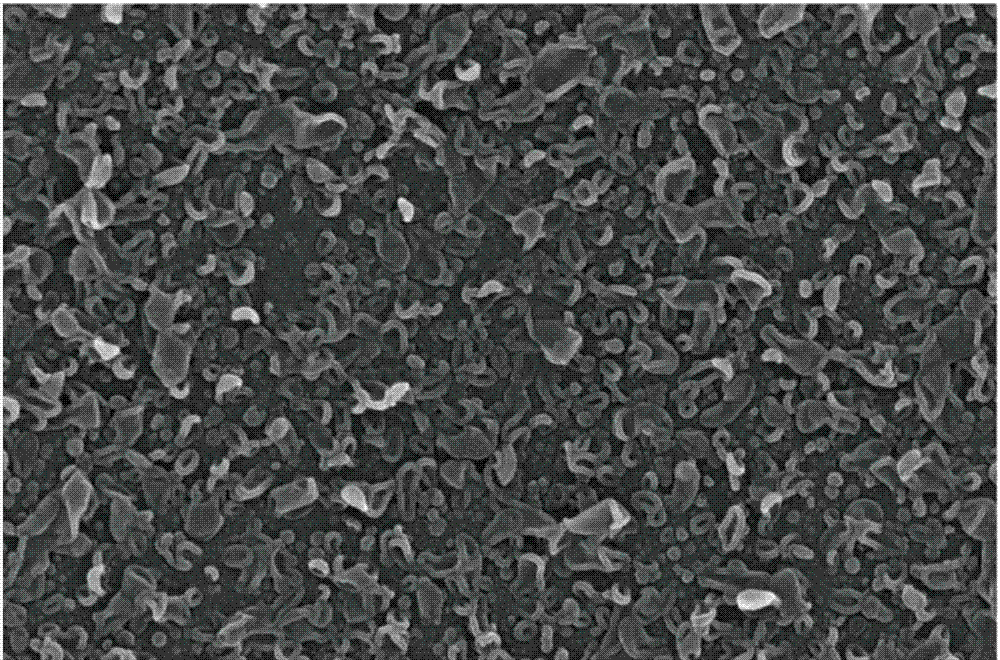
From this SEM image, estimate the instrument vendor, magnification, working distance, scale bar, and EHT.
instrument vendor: FEI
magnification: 80 K X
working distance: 5.9 mm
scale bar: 2000 nm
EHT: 15 kV